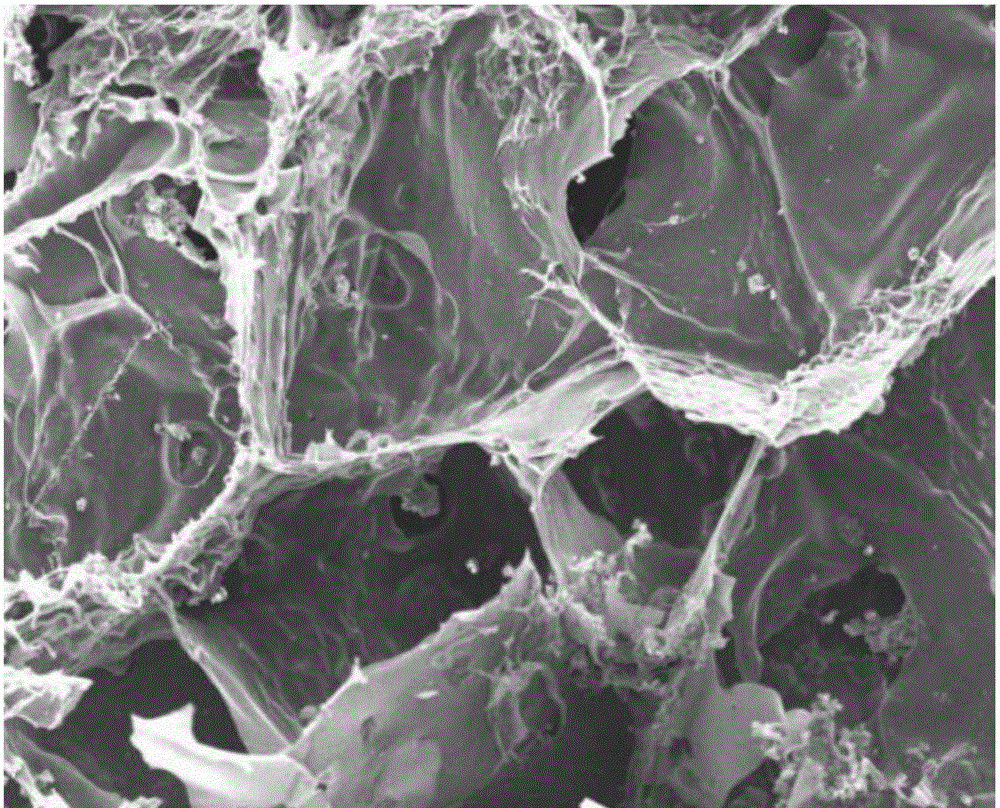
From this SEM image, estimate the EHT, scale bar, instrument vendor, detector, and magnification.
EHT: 20 kV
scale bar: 100000 nm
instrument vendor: JEOL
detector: SED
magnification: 0.1 K X